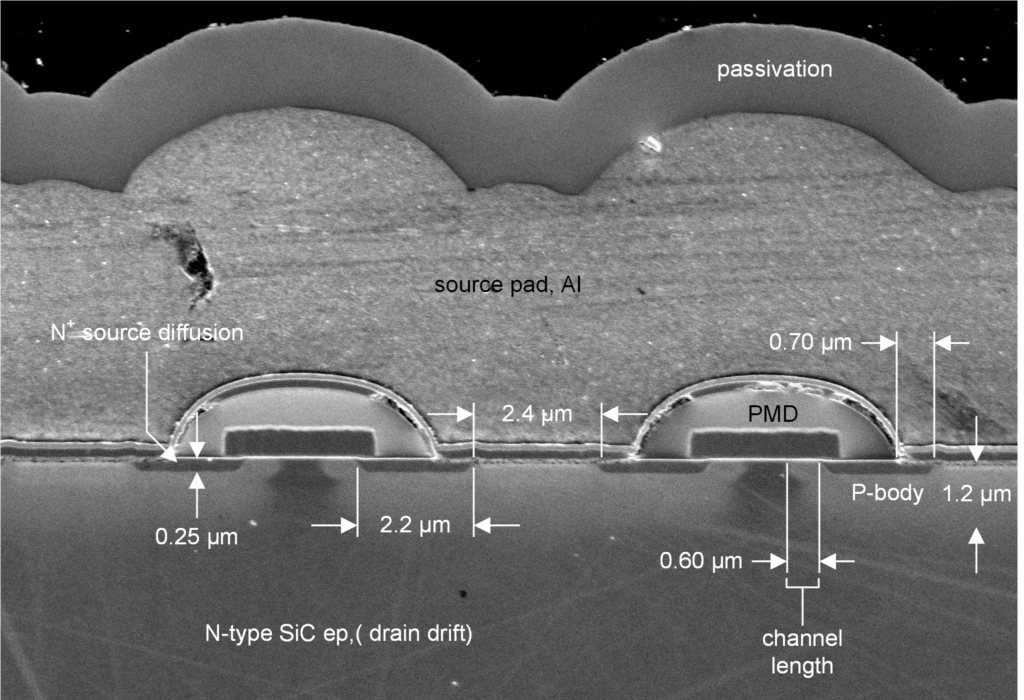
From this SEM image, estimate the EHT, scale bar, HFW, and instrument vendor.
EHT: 1 kV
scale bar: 1000 nm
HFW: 18.96 µm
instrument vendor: Zeiss